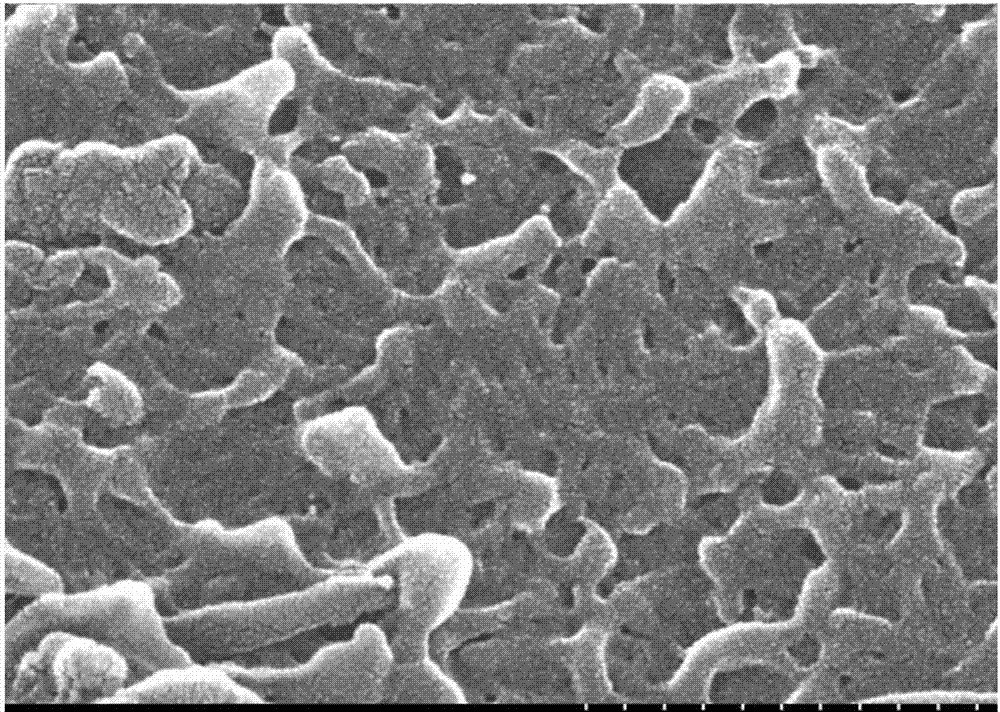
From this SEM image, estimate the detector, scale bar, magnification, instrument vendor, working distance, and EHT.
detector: SE(M)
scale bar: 50000 nm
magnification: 1 K X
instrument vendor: Hitachi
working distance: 15 mm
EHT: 15 kV